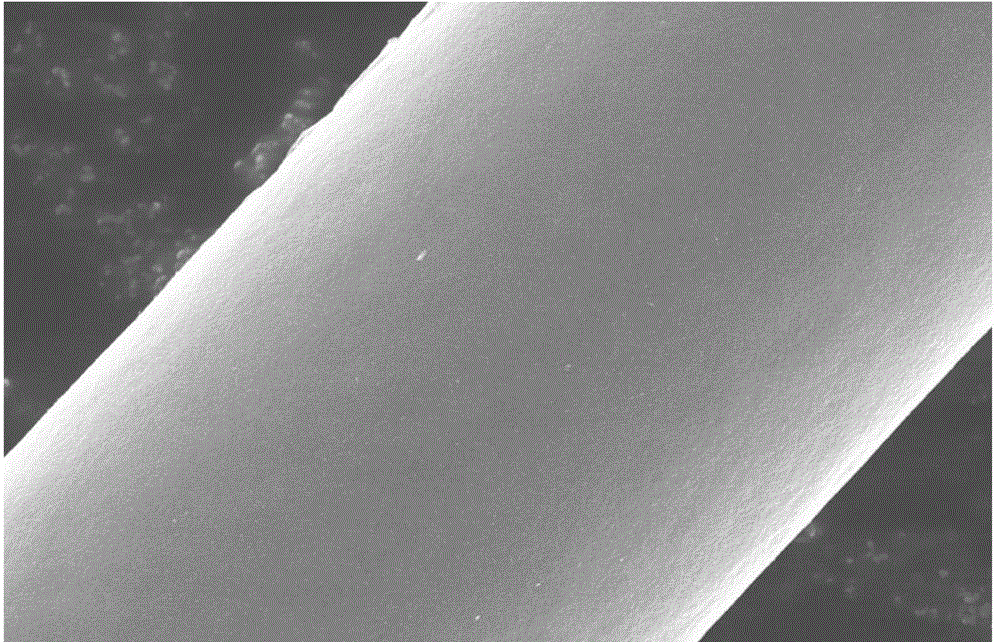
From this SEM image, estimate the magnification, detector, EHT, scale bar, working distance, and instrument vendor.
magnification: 8 K X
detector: SE(M)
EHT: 10 kV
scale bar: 5000 nm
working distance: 9 mm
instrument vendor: Hitachi